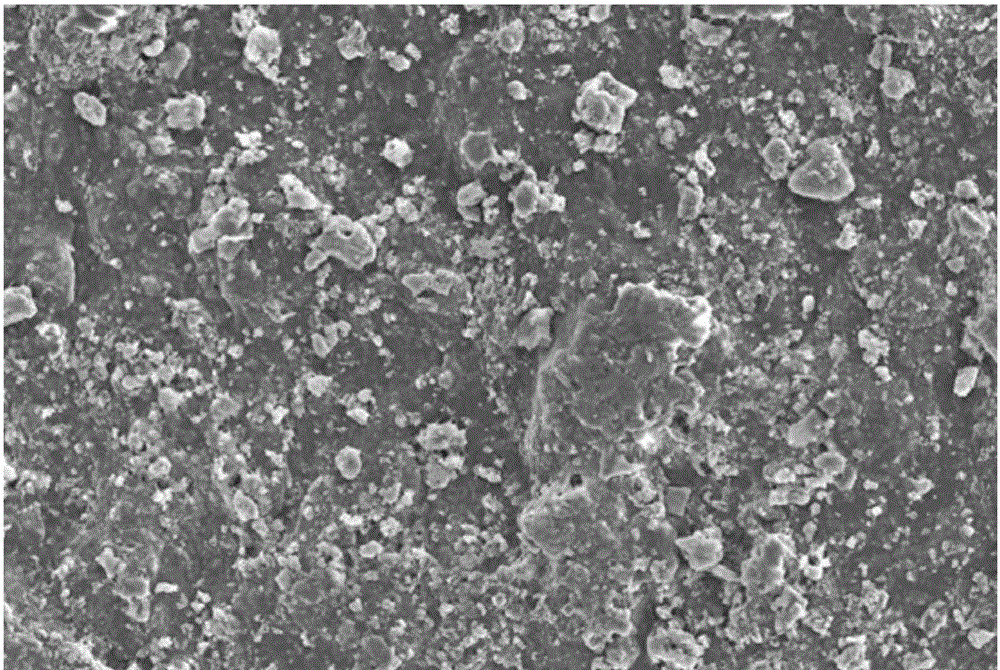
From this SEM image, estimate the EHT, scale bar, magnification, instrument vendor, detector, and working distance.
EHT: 15 kV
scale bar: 20000 nm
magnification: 0.201 K X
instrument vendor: Zeiss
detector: SE2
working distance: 11.8 mm